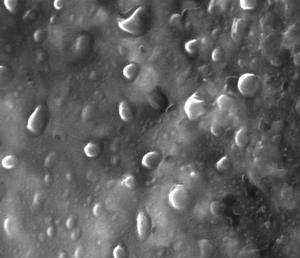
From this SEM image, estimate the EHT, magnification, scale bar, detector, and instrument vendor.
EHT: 20.03 kV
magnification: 25.6 K X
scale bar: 200 nm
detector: SE2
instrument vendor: Zeiss